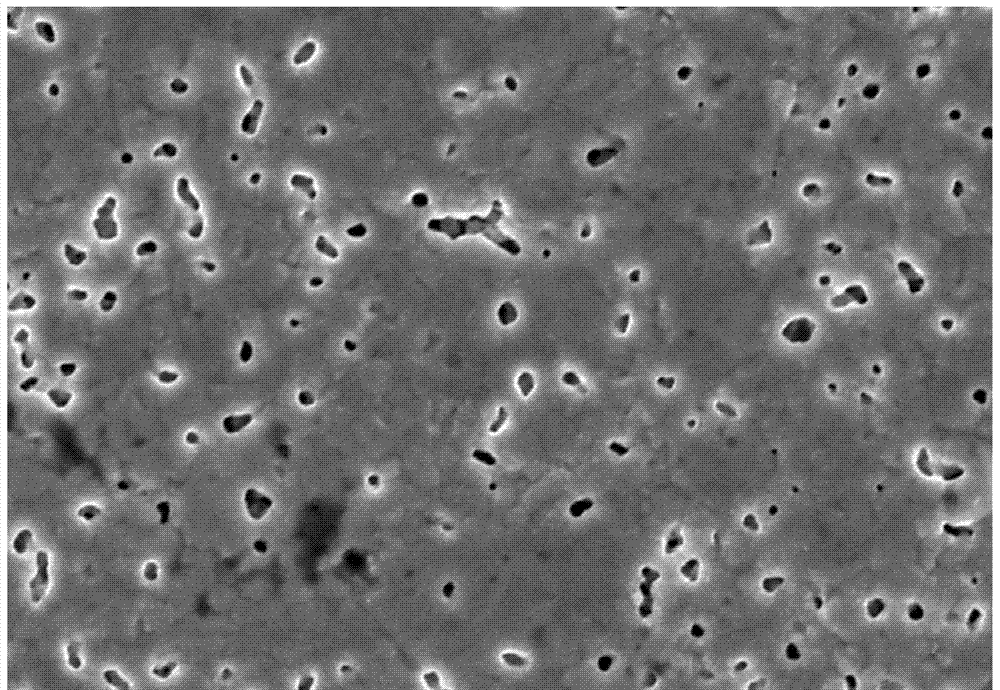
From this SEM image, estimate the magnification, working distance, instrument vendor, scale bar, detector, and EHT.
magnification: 5 K X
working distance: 9.5 mm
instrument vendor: Hitachi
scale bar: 10000 nm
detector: SE(U)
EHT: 15 kV